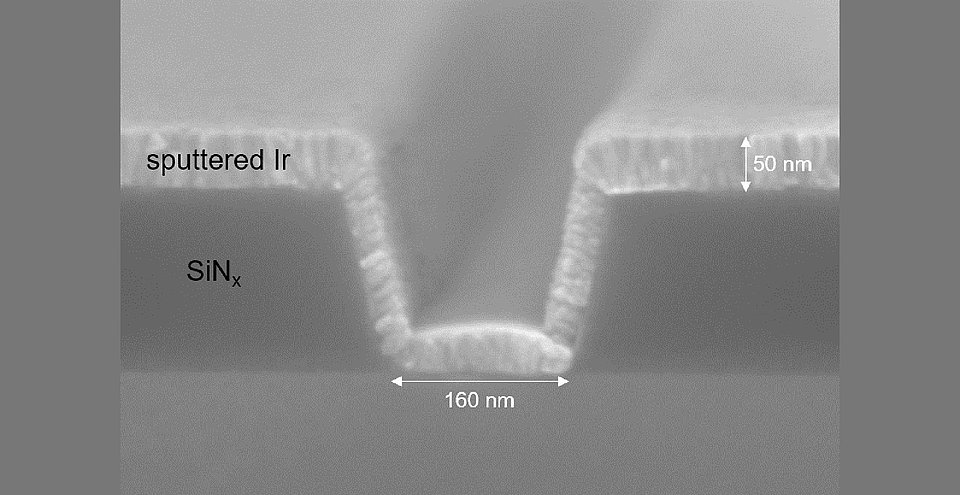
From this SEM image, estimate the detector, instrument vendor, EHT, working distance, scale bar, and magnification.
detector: SE(UL)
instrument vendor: Hitachi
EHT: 10 kV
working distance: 8.5 mm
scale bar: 200 nm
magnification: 200 K X